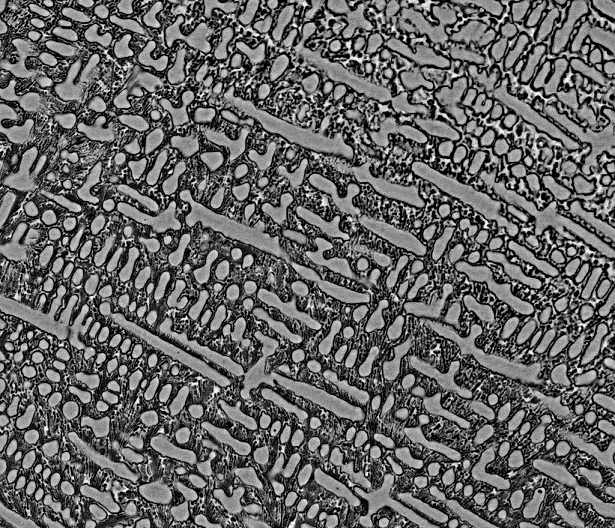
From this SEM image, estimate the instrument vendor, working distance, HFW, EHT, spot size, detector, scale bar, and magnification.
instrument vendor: FEI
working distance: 11.9 mm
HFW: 74.6 µm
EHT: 20 kV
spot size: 5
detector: BSED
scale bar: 20000 nm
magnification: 2 K X